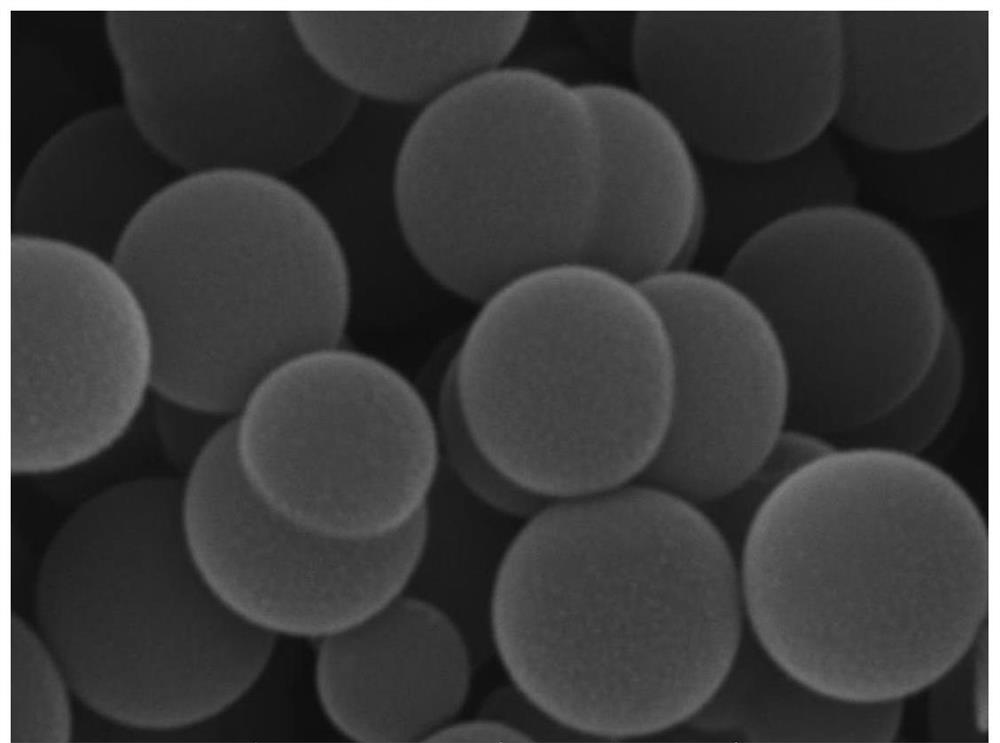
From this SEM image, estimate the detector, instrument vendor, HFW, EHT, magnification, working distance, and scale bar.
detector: SE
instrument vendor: Tescan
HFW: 4.15 µm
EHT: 30 kV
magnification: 50 K X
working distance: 7.89 mm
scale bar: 1000 nm